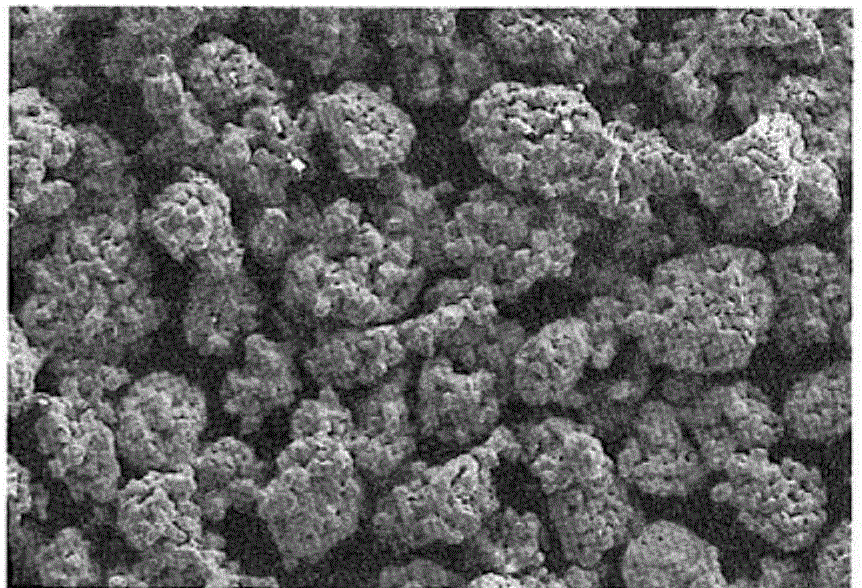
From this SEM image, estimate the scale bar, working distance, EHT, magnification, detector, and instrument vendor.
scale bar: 20000 nm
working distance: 12.8 mm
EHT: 20 kV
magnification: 0.2 K X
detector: SE2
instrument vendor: Zeiss